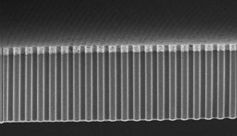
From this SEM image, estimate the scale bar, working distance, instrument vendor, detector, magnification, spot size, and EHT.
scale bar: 20000 nm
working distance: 4.9 mm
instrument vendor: FEI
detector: TLD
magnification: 1.026 K X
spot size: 3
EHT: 5 kV